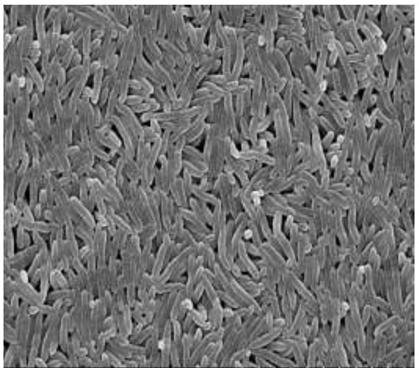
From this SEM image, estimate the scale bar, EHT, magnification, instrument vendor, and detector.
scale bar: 10000 nm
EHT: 15 kV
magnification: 5 K X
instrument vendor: Hitachi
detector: SE